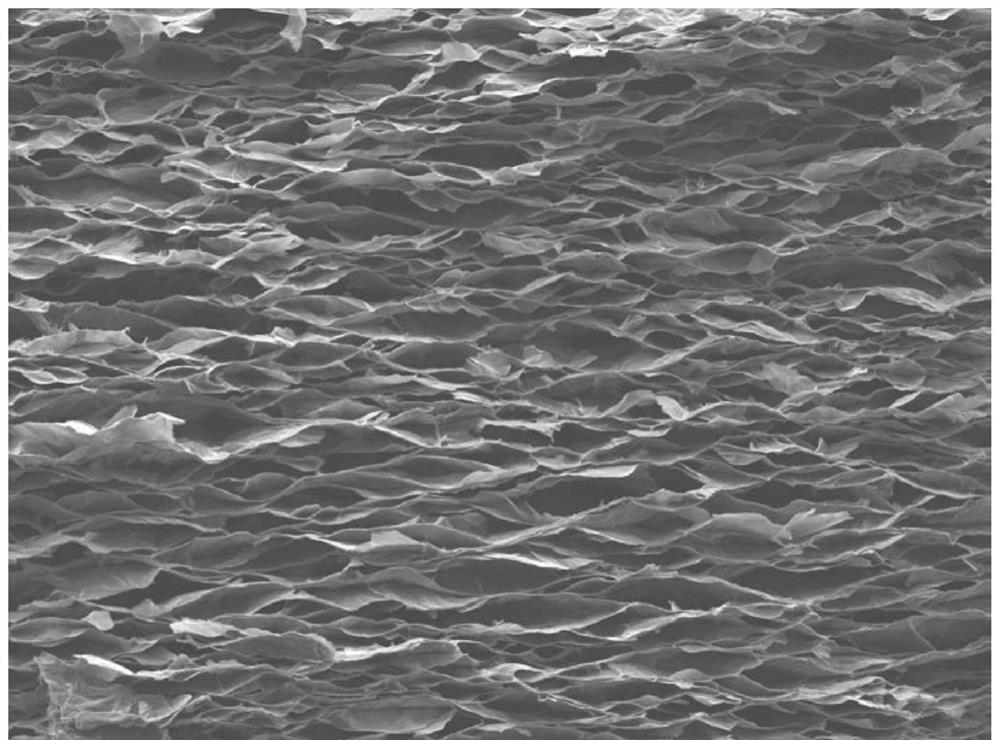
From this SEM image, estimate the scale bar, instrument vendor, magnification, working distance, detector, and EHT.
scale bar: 100000 nm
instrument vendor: JEOL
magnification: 0.1 K X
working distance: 8 mm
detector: SEI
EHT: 10 kV